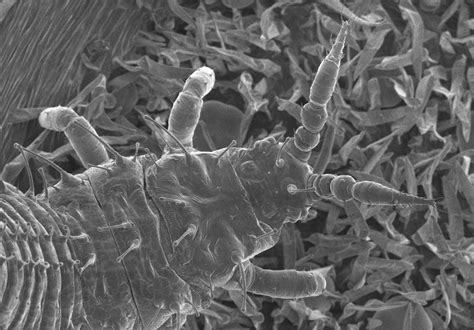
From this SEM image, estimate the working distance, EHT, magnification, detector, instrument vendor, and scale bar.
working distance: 10.2 mm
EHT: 1 kV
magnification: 0.32 K X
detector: SE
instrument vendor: Hitachi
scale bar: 100000 nm